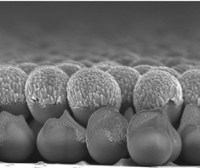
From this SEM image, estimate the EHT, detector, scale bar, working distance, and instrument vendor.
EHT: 3 kV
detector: InLens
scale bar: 200 nm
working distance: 3 mm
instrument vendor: Zeiss